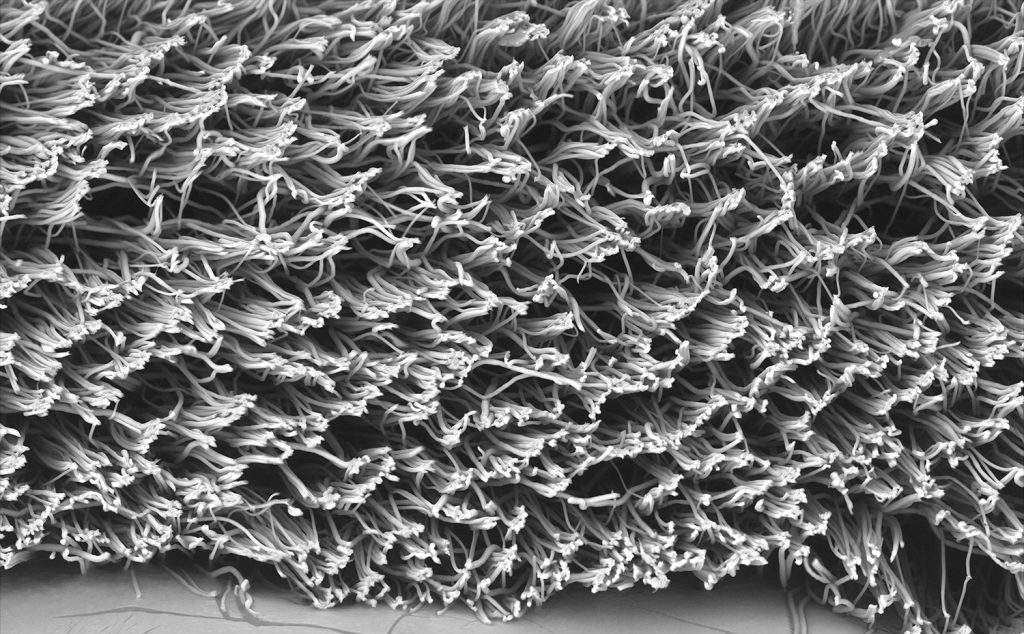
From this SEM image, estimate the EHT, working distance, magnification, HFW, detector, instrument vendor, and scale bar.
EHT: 15 kV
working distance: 6.033 mm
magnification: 1 K X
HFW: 519 µm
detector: BSD Full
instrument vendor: other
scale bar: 150000 nm